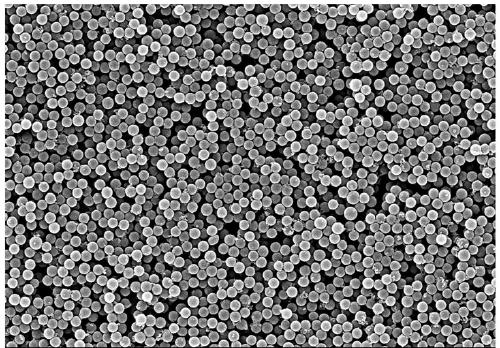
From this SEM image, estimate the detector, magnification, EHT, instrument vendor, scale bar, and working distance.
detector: SE(M)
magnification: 10 K X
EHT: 10 kV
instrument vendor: Hitachi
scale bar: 5000 nm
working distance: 7.4 mm